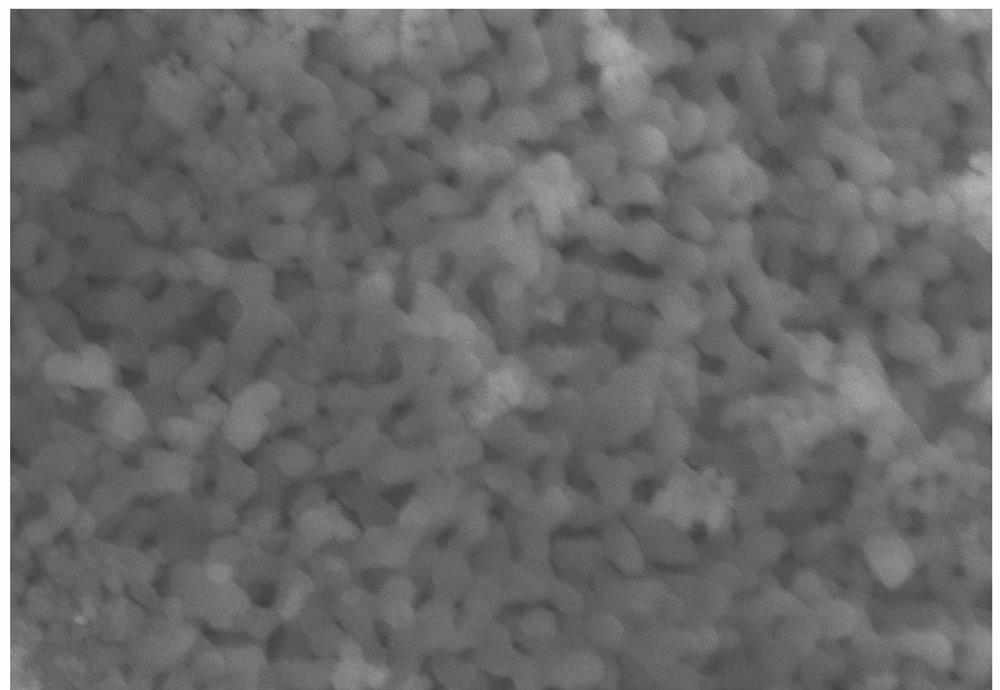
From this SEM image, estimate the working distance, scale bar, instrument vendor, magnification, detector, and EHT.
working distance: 9.2 mm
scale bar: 200 nm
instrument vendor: Zeiss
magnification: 40 K X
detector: InLens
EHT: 10 kV